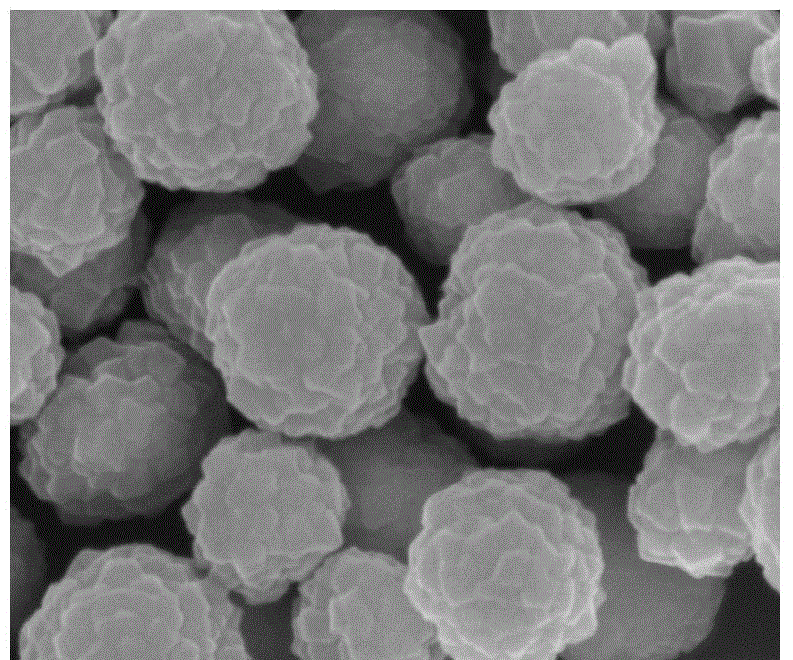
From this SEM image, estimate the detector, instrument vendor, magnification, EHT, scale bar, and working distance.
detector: TLD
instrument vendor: FEI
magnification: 200 K X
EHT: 5 kV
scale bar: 300 nm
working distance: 4.4 mm